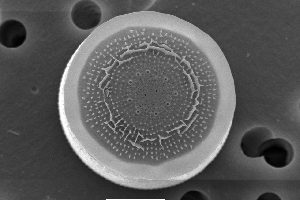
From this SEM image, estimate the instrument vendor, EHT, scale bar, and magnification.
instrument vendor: JEOL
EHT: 10 kV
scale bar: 5000 nm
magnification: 5 K X